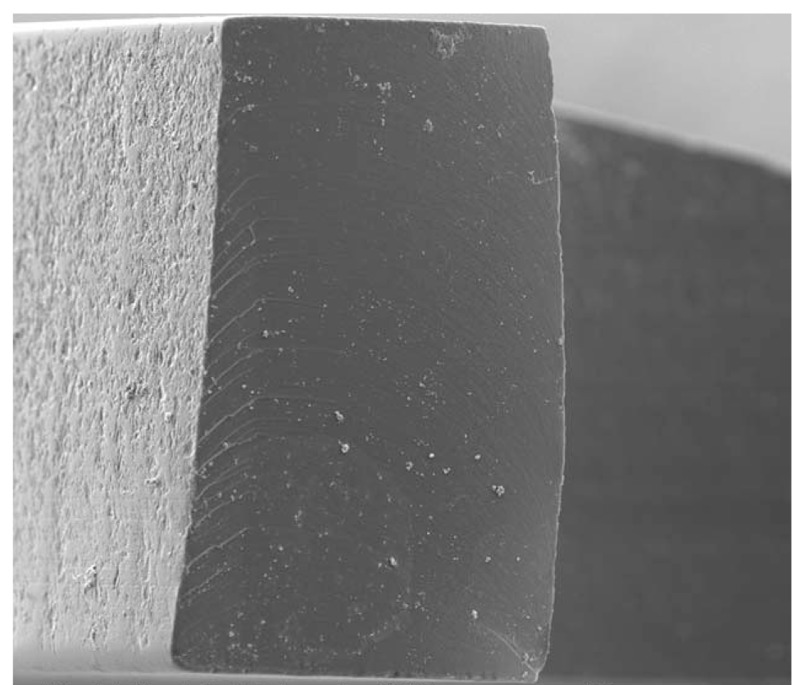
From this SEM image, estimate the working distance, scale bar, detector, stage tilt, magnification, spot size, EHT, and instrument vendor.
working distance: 9.5 mm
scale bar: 100000 nm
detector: ETD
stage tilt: -1°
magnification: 0.7 K X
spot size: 3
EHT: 10 kV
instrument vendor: FEI